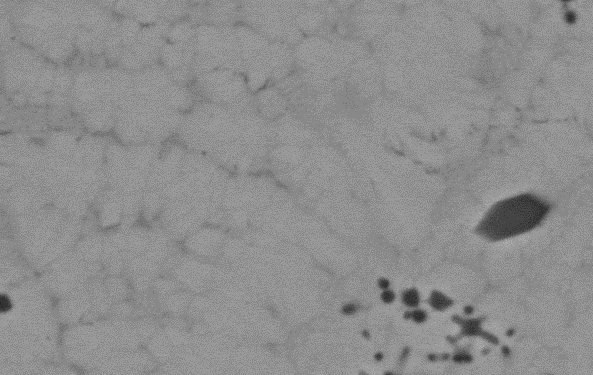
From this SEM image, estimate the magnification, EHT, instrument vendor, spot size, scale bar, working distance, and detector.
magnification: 5 K X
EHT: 20 kV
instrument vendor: FEI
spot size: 4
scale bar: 5000 nm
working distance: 19.9 mm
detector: BSE